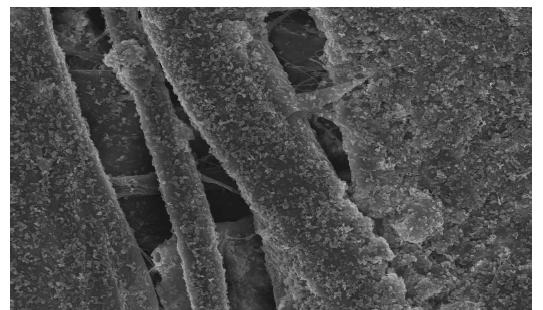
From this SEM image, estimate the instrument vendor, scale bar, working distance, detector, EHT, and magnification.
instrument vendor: Hitachi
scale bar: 50000 nm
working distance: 12 mm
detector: SE(U)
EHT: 15 kV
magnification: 1 K X